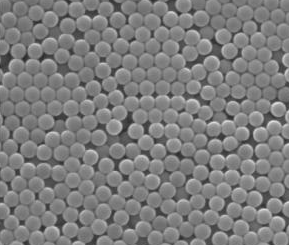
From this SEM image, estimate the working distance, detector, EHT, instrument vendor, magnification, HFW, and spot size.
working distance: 9.8 mm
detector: SE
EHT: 30 kV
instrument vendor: FEI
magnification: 25 K X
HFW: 16.6 µm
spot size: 4.5